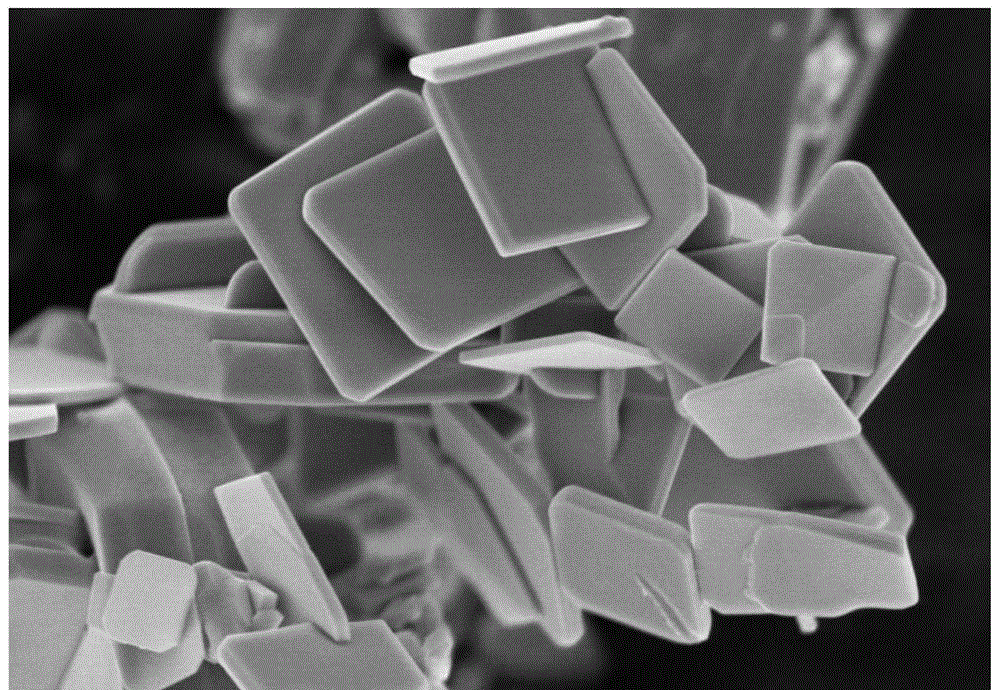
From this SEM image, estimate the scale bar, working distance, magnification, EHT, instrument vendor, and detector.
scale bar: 1000 nm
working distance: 3.1 mm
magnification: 30 K X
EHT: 3 kV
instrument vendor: Hitachi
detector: SE(M,LA0)